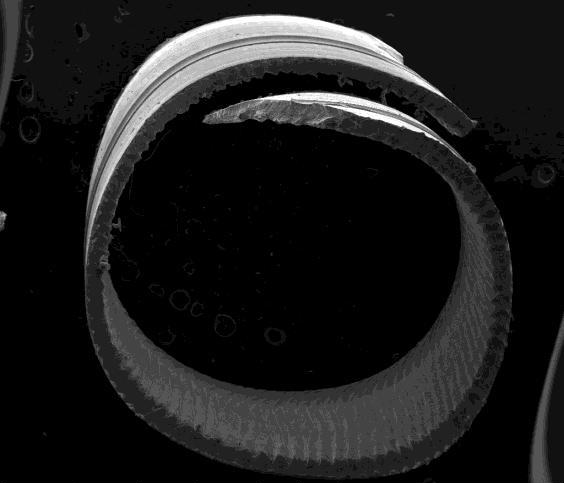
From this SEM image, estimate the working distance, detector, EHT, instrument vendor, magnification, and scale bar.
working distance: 13.6 mm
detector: ETD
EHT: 20 kV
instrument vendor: FEI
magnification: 0.042 K X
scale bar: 2000000 nm